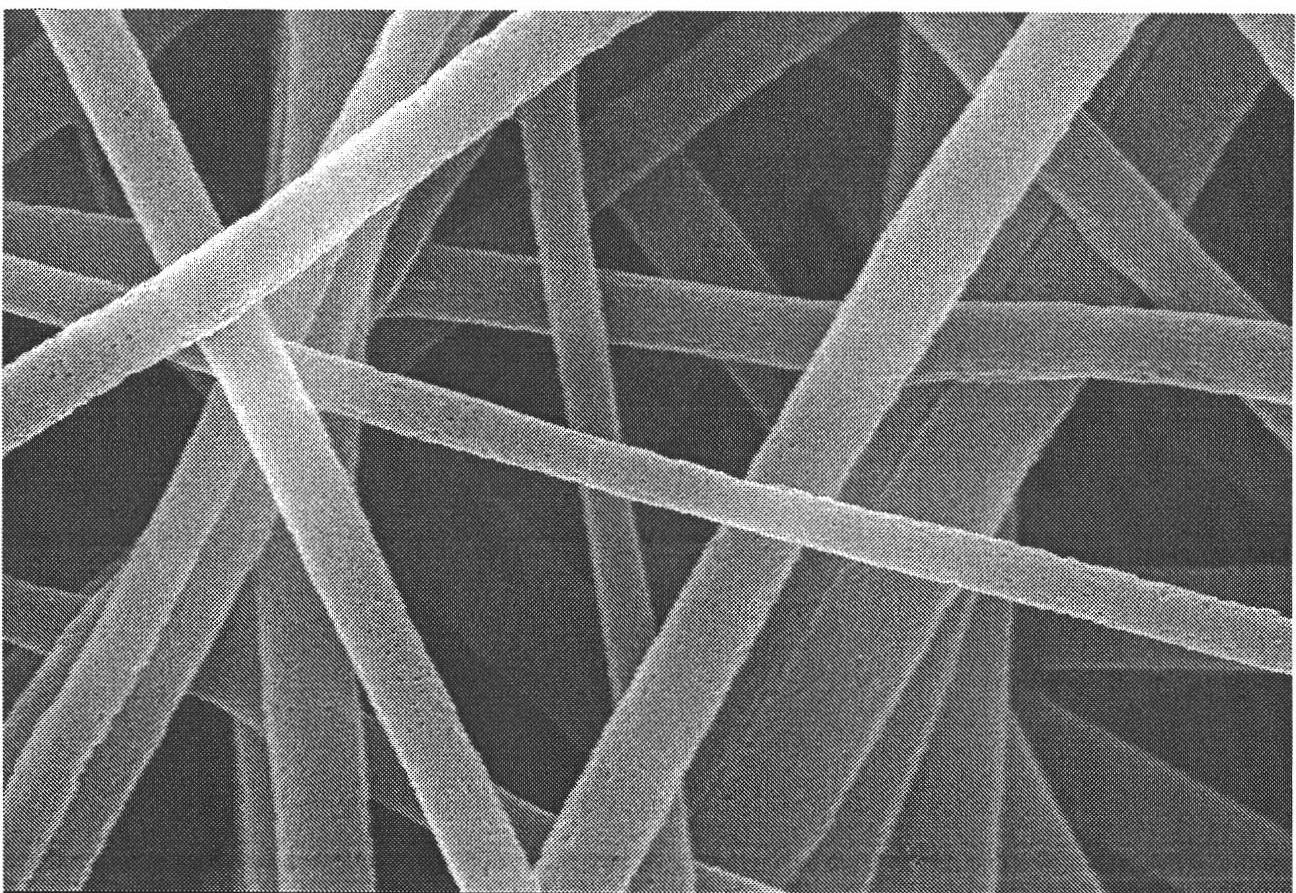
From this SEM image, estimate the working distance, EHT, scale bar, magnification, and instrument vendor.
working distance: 11.7 mm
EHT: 20 kV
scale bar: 1000 nm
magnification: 30 K X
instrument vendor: Hitachi